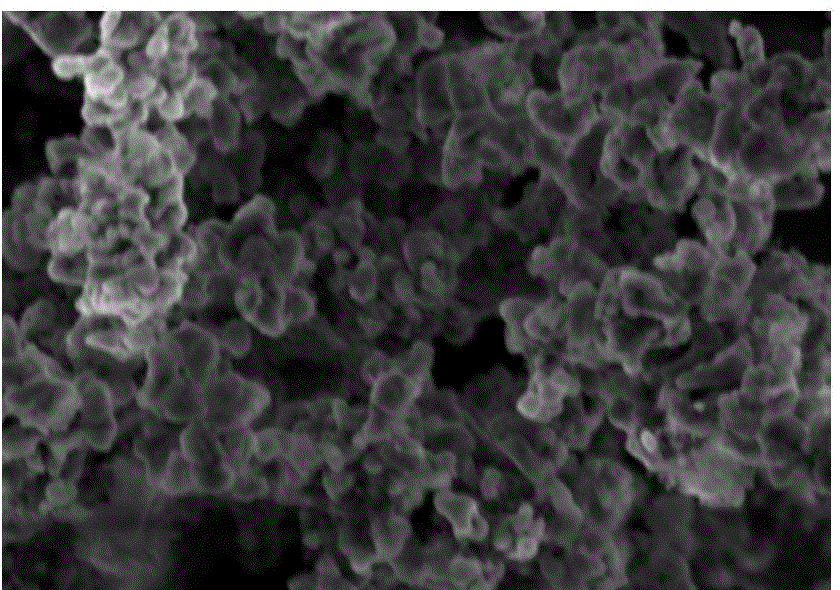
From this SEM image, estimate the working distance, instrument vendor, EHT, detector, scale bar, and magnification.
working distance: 8.3 mm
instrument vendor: Hitachi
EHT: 3 kV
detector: SE(M)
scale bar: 1000 nm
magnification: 30 K X